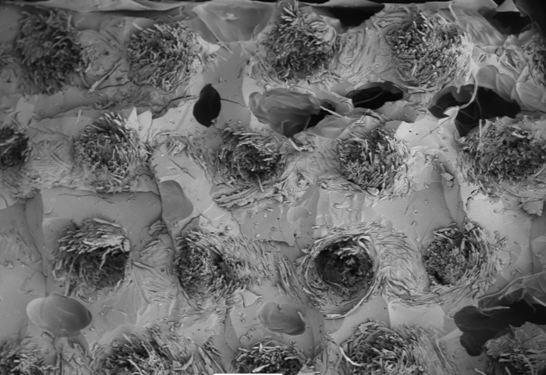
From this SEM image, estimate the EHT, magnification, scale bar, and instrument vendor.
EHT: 15 kV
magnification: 0.033 K X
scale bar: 500000 nm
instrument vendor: JEOL